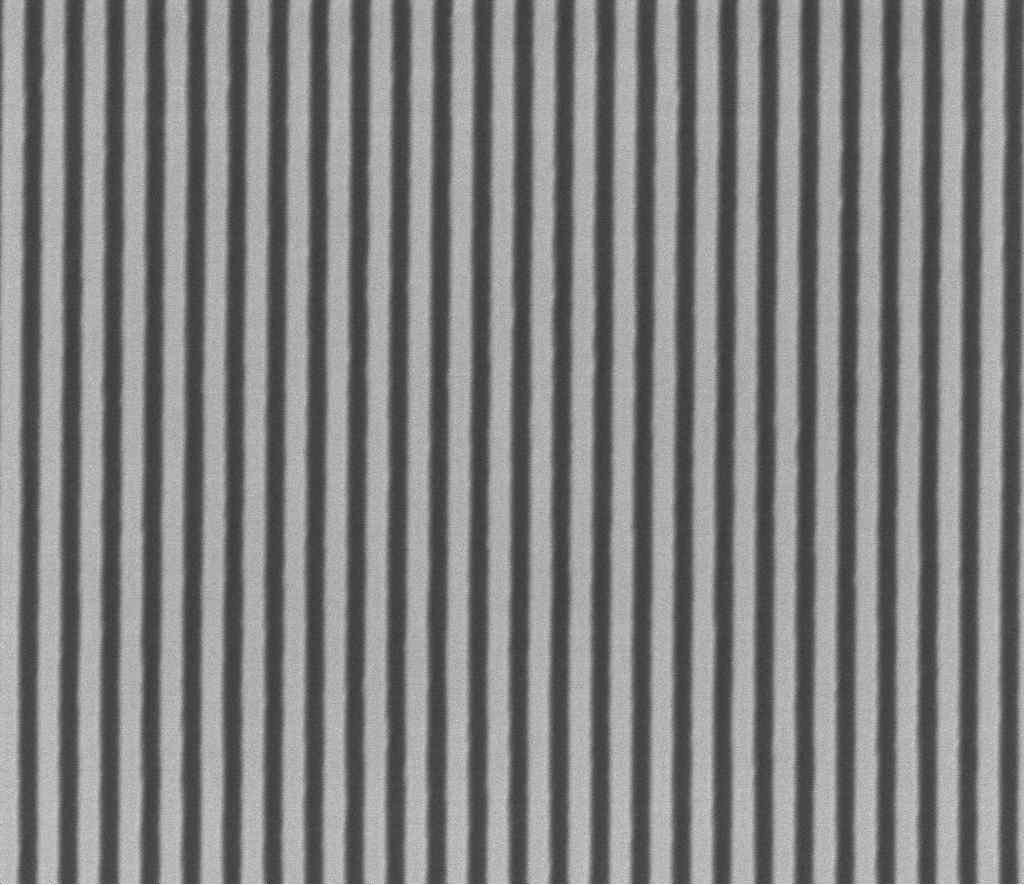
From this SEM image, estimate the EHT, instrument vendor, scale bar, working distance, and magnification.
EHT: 5 kV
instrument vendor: FEI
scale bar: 3000 nm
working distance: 3.7 mm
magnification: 25 K X